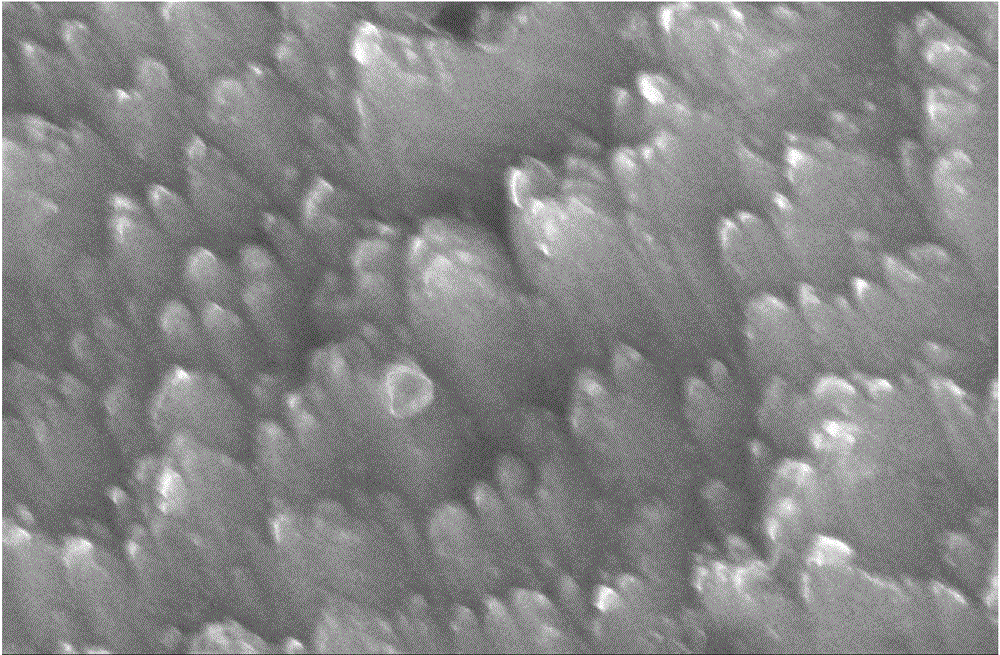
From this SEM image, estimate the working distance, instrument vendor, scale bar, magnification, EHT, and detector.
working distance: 9.7 mm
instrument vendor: Hitachi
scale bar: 1000 nm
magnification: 50 K X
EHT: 15 kV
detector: SE(M)